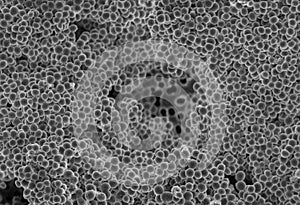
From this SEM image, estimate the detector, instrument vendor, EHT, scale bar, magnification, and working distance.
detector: SE(UL)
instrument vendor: Hitachi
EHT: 5 kV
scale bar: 10000 nm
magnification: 4.5 K X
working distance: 9.4 mm